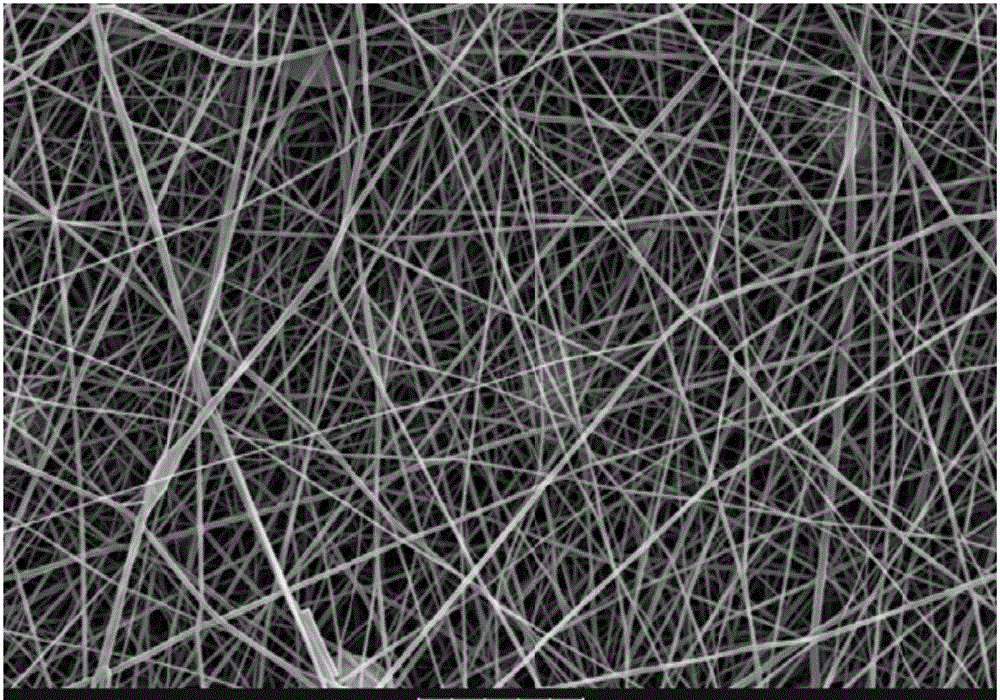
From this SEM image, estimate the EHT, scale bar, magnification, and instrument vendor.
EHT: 20 kV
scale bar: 10000 nm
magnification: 2 K X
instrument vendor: other